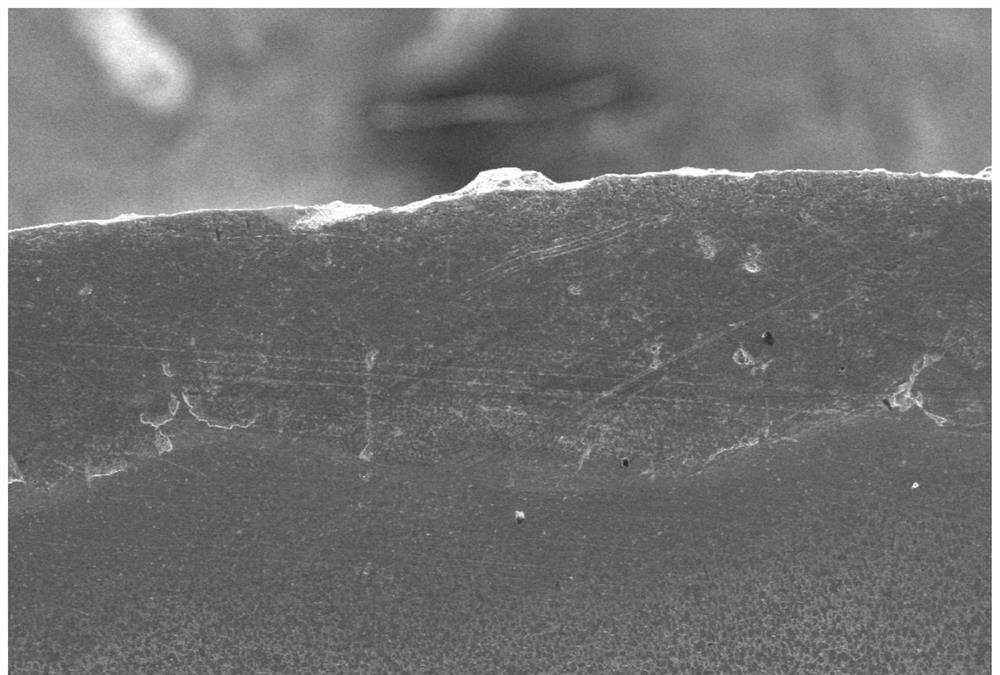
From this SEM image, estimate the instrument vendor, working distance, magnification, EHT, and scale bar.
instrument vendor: JEOL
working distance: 8 mm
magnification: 0.05 K X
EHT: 15 kV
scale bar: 500000 nm